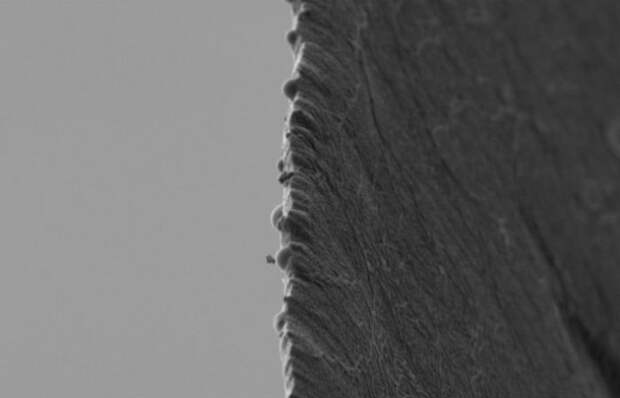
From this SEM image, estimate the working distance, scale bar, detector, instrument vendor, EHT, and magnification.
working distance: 6.1 mm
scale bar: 1000 nm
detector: SE2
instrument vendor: Zeiss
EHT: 1 kV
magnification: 10 K X